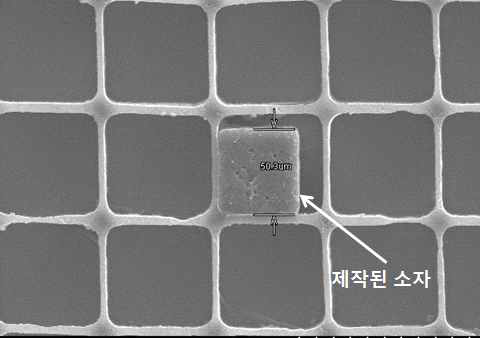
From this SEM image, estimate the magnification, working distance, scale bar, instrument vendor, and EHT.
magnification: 0.45 K X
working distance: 10.5 mm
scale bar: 100000 nm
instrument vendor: Hitachi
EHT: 25 kV